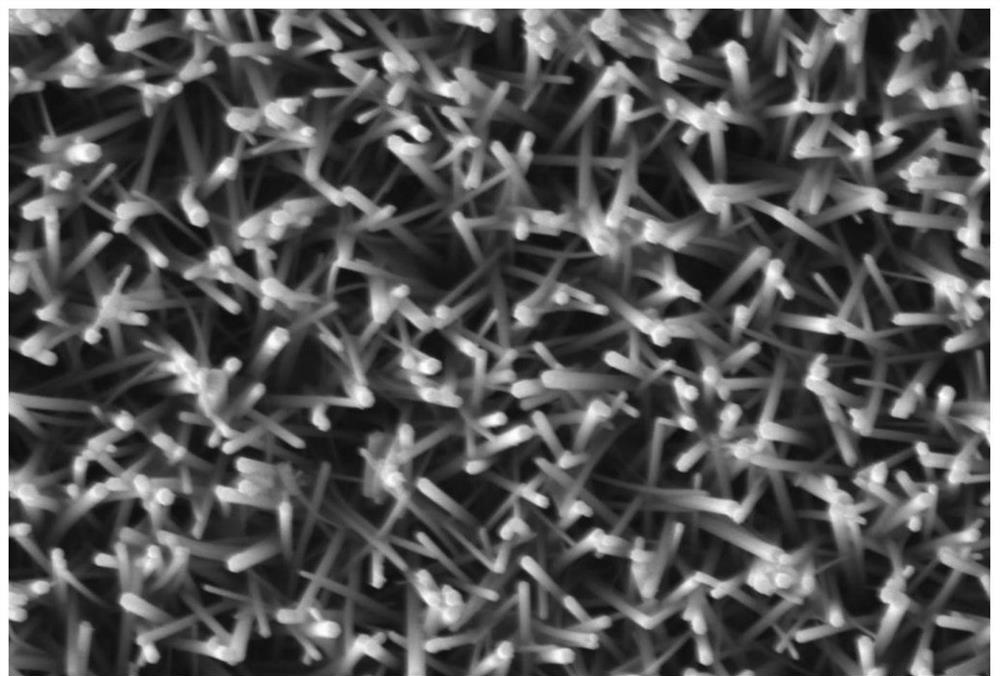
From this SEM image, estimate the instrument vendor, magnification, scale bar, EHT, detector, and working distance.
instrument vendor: Zeiss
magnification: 50 K X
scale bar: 200 nm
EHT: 5 kV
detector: SE2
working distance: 11.8 mm